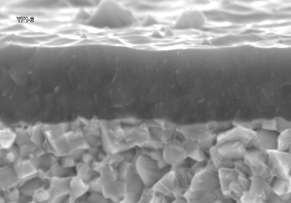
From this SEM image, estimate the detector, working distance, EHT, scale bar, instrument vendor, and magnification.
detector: SE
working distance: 6.8 mm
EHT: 15 kV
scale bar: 3000 nm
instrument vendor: Hitachi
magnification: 15 K X